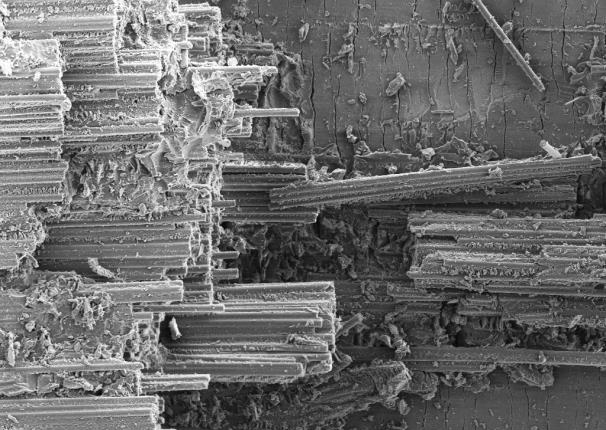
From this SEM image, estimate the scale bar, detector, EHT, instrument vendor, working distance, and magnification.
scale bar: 100000 nm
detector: SE2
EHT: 7 kV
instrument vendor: Zeiss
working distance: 10.4 mm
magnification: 0.5 K X